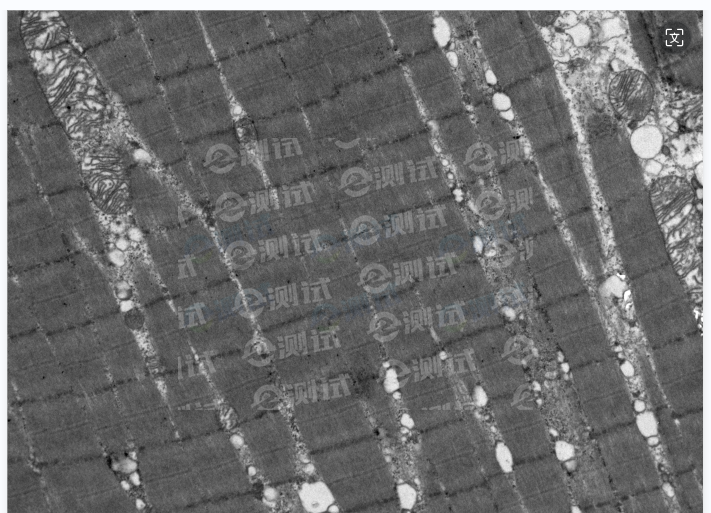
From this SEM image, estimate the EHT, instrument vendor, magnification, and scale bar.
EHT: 80 kV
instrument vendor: Hitachi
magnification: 3 K X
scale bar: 2000 nm